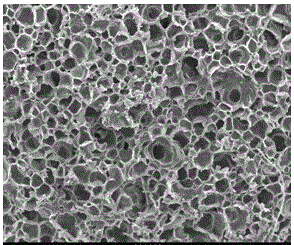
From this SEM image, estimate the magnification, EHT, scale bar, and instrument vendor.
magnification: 0.1 K X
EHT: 25 kV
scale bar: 100000 nm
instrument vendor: other